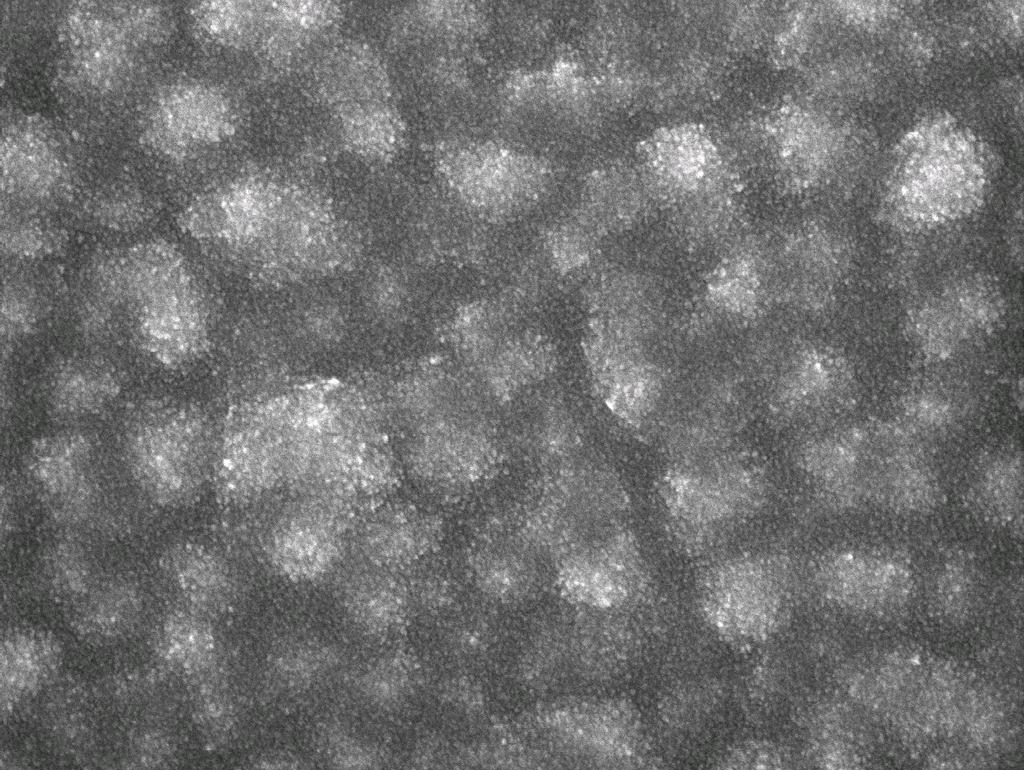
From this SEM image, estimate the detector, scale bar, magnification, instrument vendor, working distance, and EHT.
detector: SEI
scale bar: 100 nm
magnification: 40 K X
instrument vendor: JEOL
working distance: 8.5 mm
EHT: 5 kV